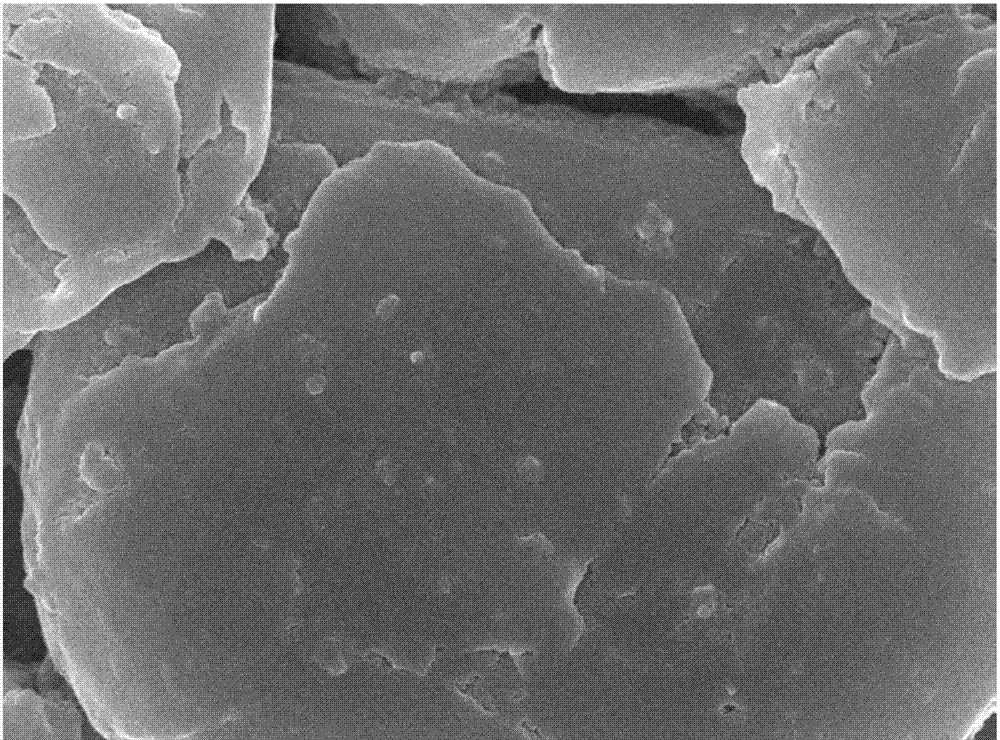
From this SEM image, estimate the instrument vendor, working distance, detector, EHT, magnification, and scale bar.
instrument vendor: JEOL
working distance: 7.5 mm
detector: SEM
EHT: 15 kV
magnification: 10 K X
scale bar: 1000 nm